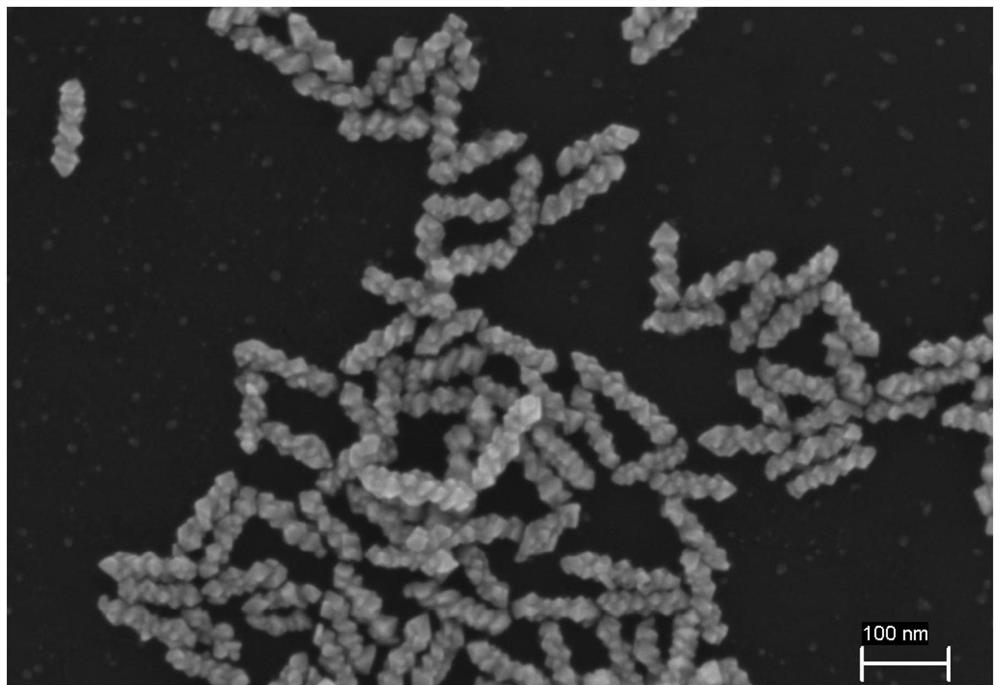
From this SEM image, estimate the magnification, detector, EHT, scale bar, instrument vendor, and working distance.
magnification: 100 K X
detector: InLens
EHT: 5 kV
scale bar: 100 nm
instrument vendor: Zeiss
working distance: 3.3 mm